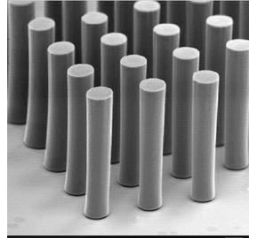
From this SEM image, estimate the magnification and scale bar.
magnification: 1 K X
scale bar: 10000 nm